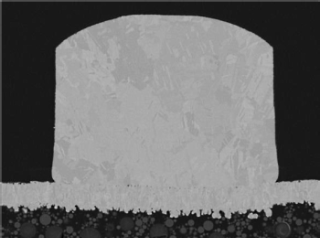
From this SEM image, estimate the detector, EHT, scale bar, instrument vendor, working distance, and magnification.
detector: BED-C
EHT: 5 kV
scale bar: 1000 nm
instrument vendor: JEOL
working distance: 10.1 mm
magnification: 3 K X